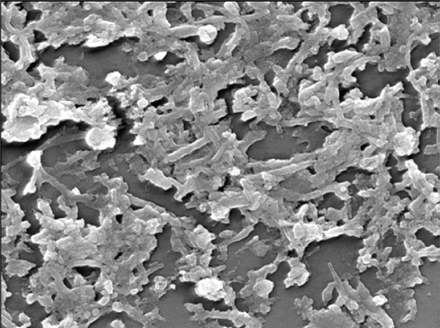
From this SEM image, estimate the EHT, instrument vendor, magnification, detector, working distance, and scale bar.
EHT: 15 kV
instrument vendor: JEOL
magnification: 20 K X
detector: SEI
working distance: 11.9 mm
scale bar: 1000 nm